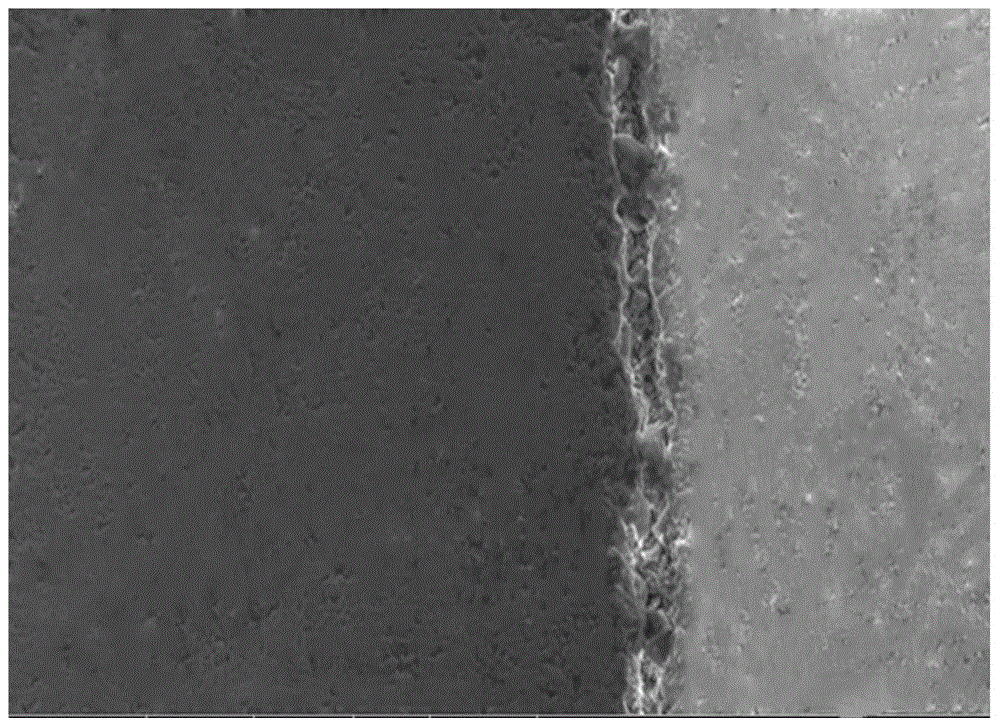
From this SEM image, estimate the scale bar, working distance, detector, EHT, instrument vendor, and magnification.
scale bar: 500000 nm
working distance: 15.6 mm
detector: SE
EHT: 30 kV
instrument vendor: FEI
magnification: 0.2 K X